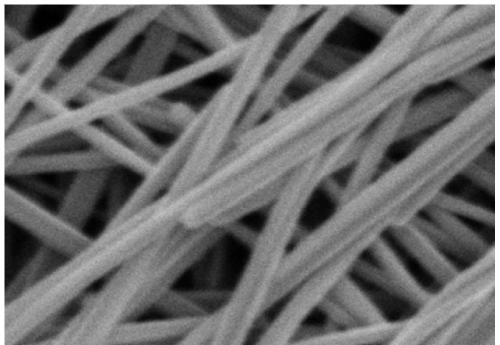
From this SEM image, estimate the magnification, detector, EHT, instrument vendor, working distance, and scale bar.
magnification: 80 K X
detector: SE(M)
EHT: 3 kV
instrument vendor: Hitachi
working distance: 9.5 mm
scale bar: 500 nm